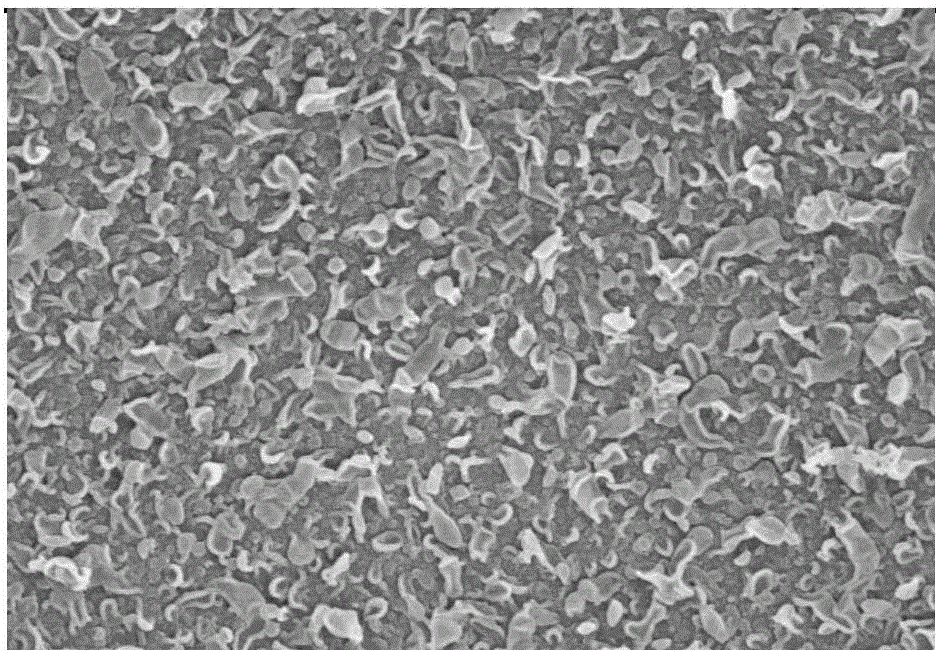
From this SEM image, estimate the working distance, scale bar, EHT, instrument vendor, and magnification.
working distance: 8.5 mm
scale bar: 2000 nm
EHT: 10 kV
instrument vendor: Hitachi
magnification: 20 K X